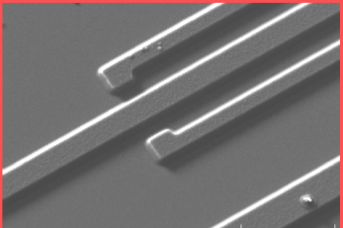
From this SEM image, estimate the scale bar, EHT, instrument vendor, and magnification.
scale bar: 10000 nm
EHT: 10 kV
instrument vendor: other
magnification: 2.5 K X